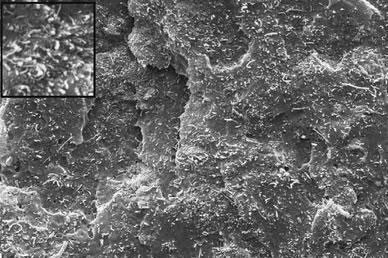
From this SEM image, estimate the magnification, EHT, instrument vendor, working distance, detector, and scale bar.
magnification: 3 K X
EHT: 15 kV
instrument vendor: Zeiss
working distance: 14 mm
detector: SE1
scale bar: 1000 nm